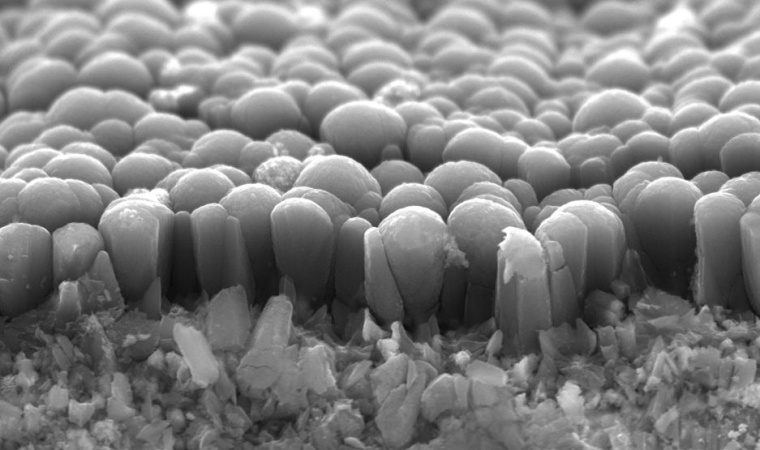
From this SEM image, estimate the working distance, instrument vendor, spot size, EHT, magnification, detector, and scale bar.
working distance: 8 mm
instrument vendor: JEOL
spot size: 30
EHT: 20 kV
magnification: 3 K X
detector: SEI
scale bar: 5000 nm